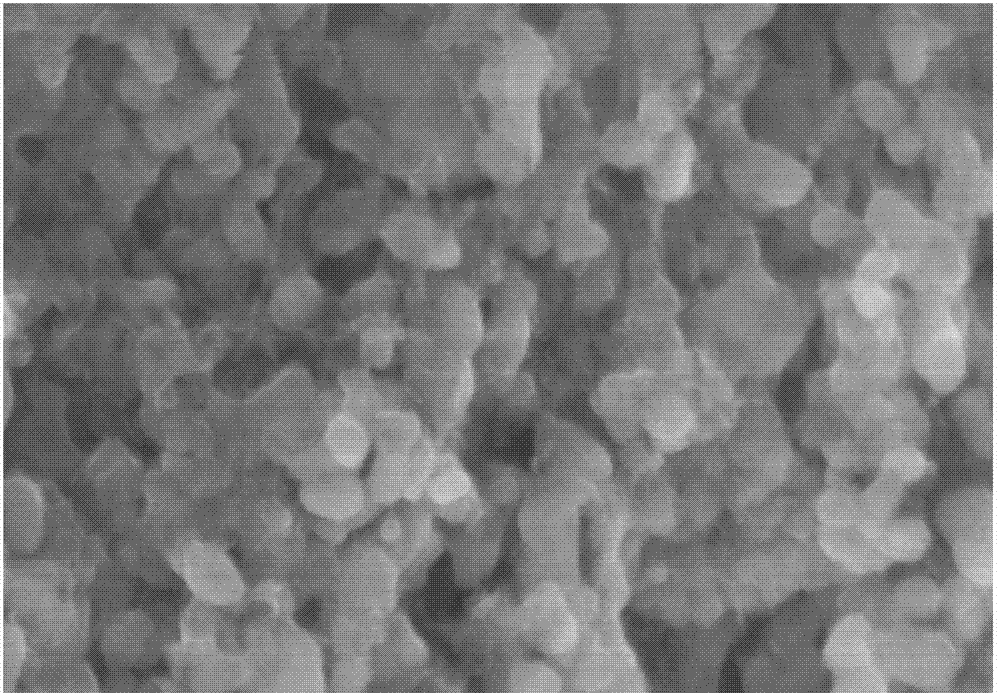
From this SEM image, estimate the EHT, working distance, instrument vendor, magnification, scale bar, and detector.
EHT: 5 kV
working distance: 8.2 mm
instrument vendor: Hitachi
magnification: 100 K X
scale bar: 500 nm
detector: SE(M)